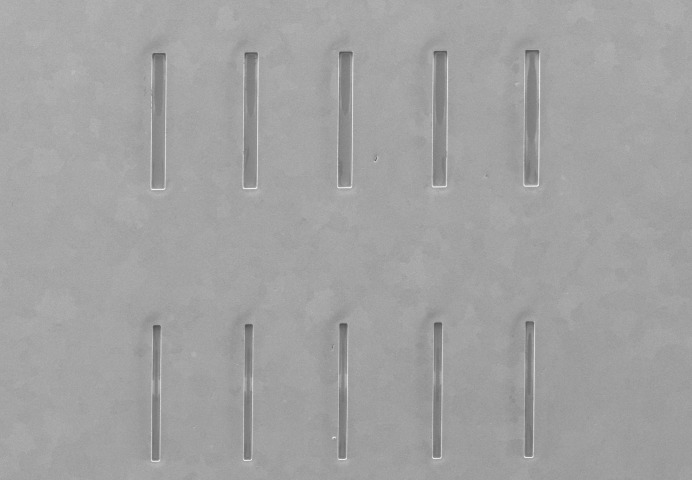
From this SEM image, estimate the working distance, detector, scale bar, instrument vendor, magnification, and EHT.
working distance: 12.2 mm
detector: SE(M)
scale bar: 1000000 nm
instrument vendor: Hitachi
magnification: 0.05 K X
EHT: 5 kV